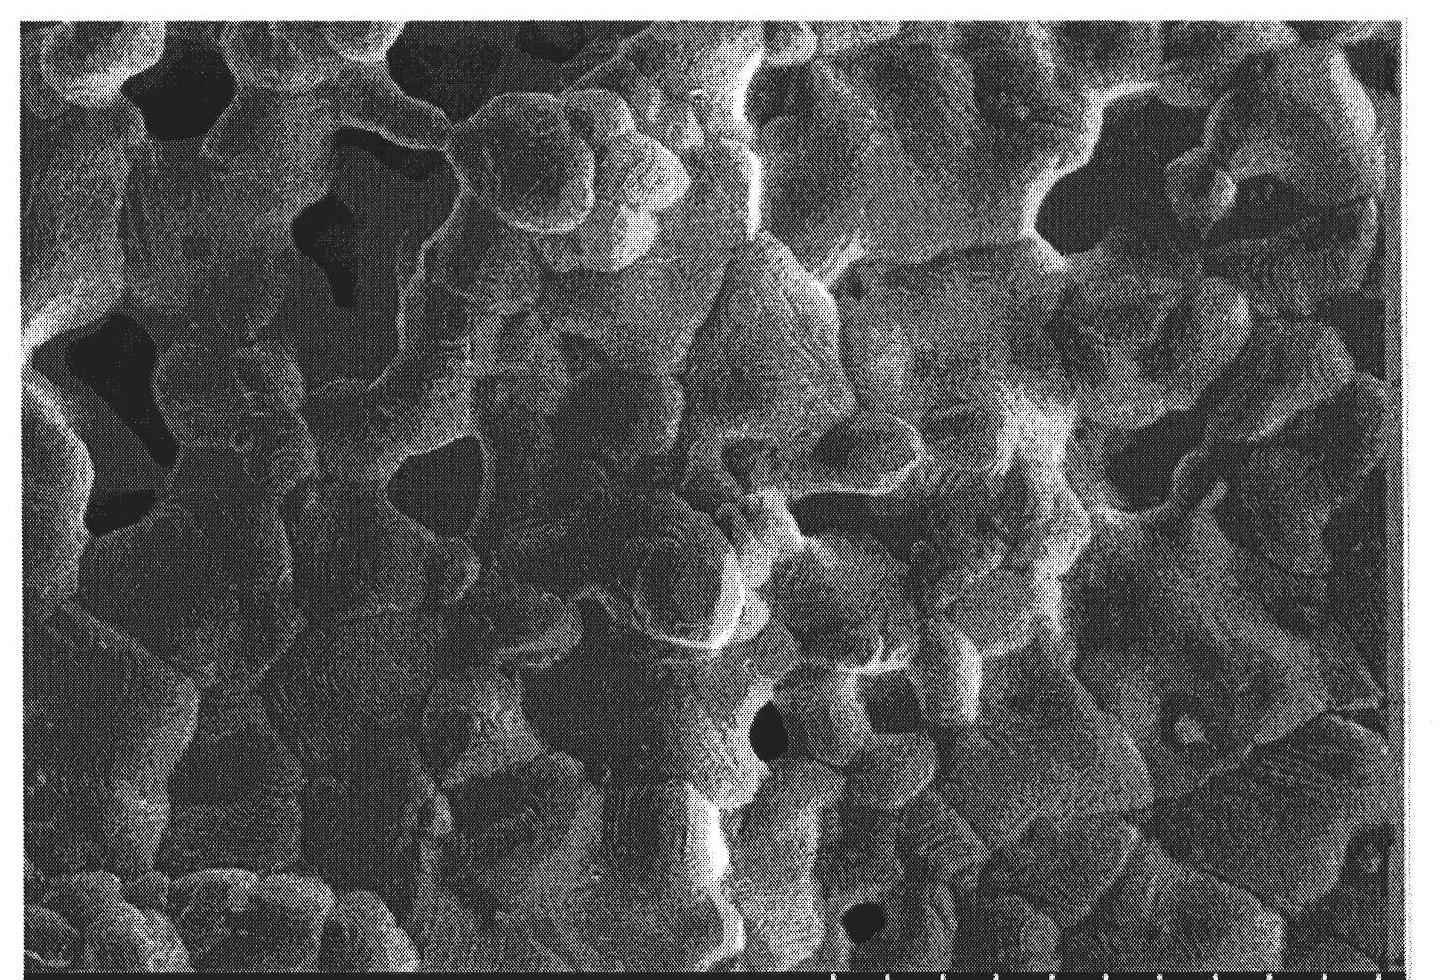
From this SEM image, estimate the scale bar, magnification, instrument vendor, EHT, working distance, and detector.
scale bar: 10000 nm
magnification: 5 K X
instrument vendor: Hitachi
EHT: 5 kV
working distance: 8.3 mm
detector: SE(M)